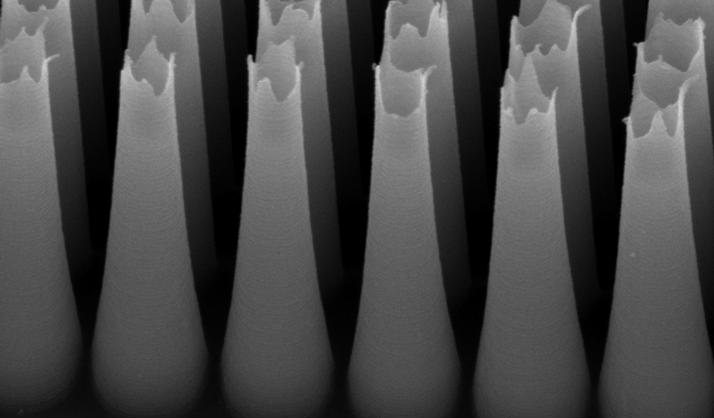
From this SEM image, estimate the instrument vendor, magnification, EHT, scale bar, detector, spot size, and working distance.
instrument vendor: FEI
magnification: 22.82 K X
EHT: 10 kV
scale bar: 1000 nm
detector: CCD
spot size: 3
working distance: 13.9 mm